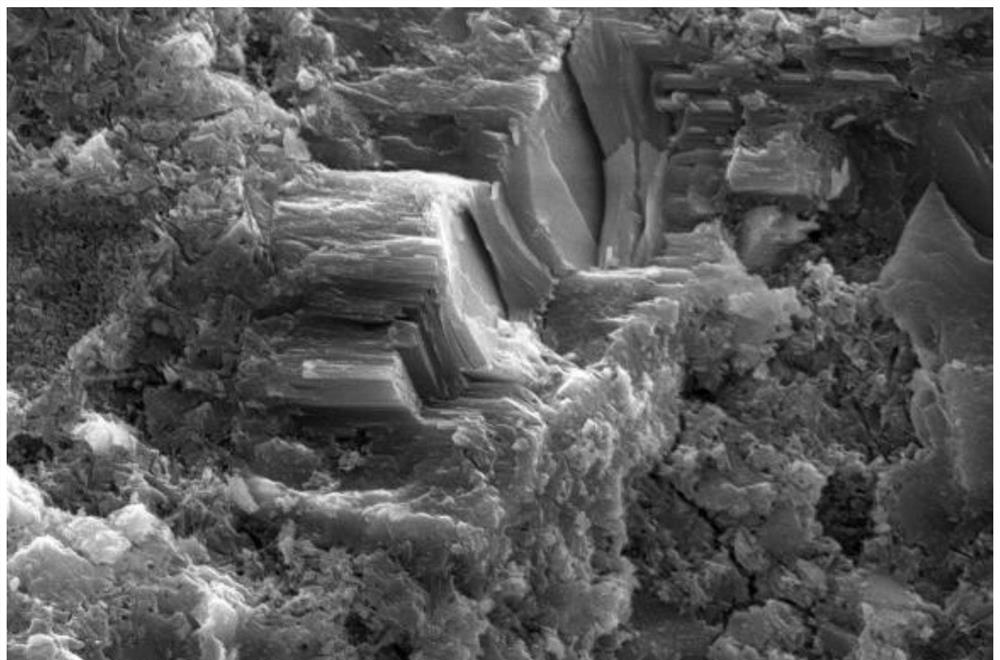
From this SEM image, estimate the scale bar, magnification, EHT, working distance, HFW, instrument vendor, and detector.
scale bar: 10000 nm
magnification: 5 K X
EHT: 15 kV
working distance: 10.1 mm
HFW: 25.4 µm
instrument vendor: FEI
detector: ETD